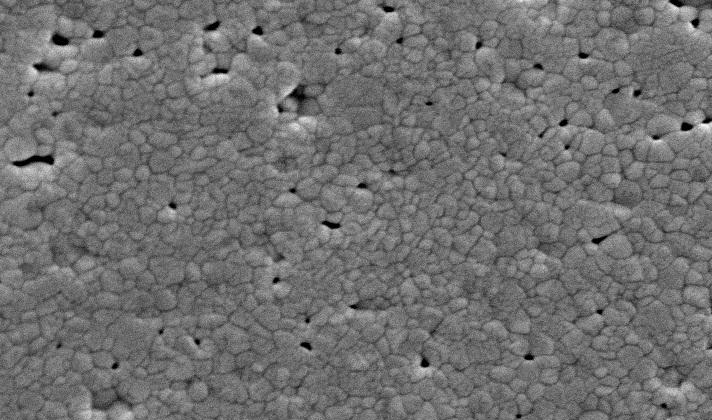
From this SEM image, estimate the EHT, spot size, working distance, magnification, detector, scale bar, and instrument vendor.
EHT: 20 kV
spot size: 3.4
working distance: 9.7 mm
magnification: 10 K X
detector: SE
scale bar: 2000 nm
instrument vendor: FEI